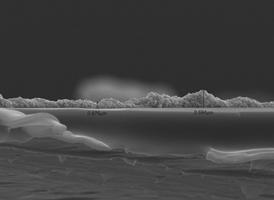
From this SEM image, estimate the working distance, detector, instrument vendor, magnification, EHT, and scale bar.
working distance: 9.8 mm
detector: SEI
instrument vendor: JEOL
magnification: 3 K X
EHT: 15 kV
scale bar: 1000 nm